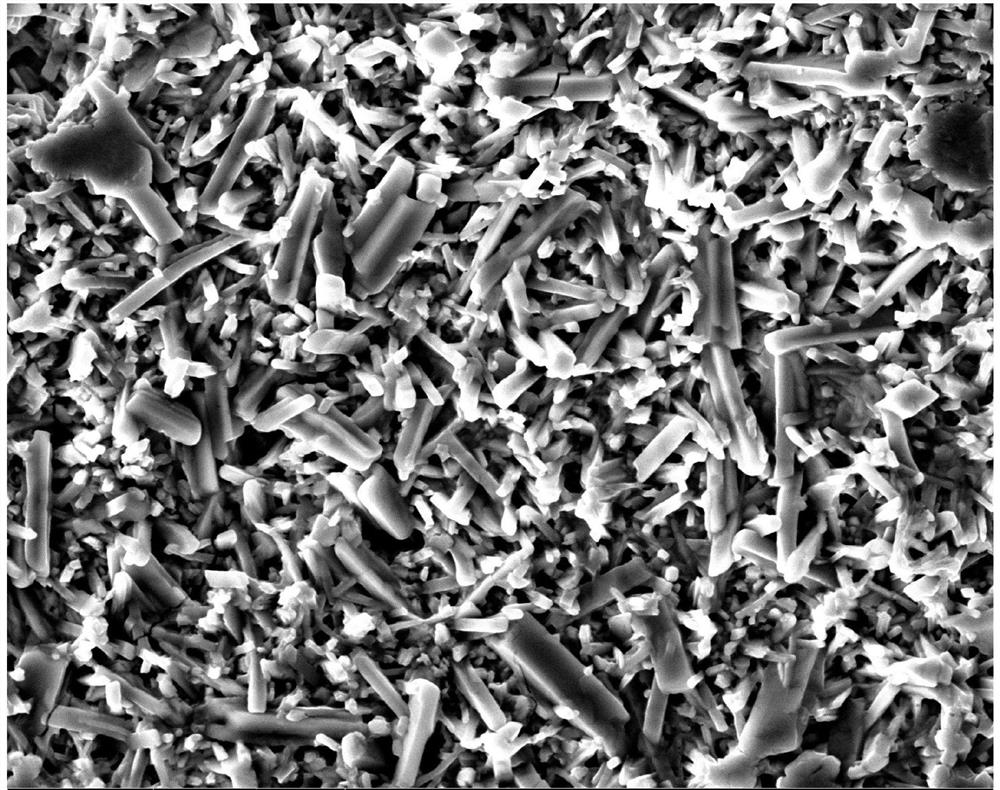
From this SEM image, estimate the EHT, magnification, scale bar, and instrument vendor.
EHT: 25 kV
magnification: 2 K X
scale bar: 40000 nm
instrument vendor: FEI